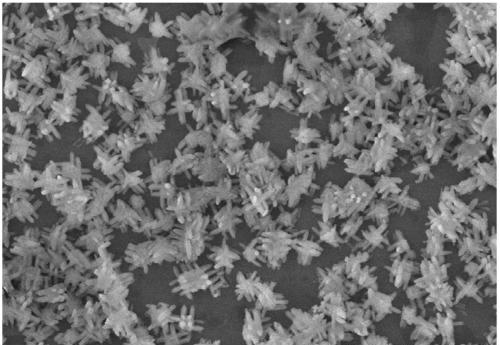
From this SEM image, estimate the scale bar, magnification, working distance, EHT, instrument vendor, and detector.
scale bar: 5000 nm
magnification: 10 K X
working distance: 8.9 mm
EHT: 5 kV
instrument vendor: Hitachi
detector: SE(UL)